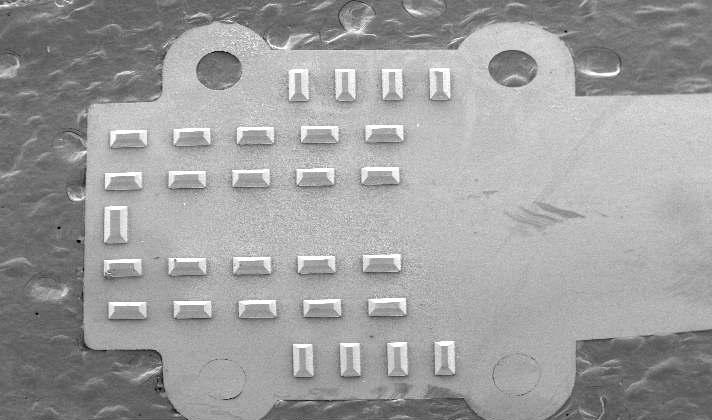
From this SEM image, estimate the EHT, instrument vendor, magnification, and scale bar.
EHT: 5 kV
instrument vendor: FEI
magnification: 0.02 K X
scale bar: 1000000 nm